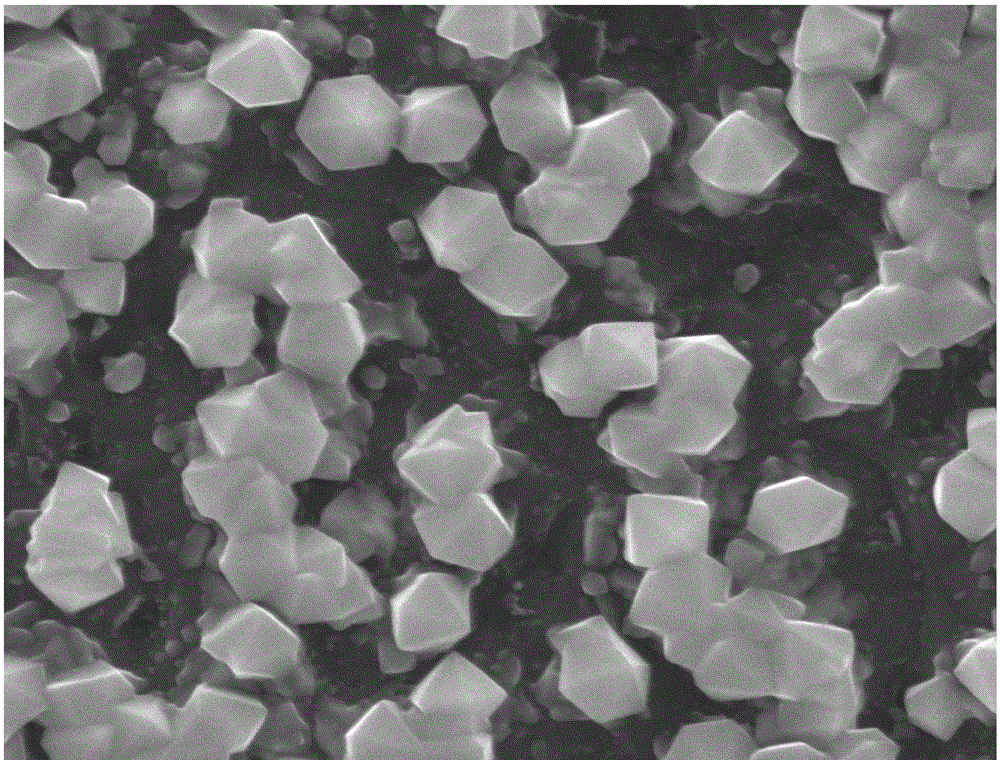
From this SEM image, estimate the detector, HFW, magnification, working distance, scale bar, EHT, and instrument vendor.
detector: ETD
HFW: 6.76 µm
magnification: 20 K X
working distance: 11.9 mm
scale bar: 1000 nm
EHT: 20 kV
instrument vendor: FEI